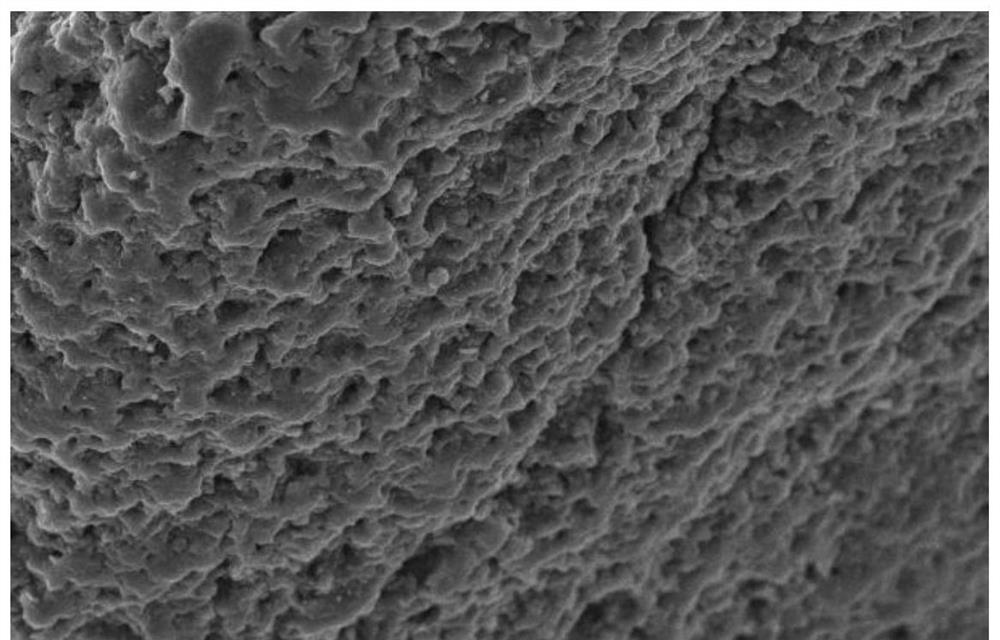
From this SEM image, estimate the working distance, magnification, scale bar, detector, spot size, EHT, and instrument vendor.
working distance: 13 mm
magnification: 0.2 K X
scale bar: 100000 nm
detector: SEI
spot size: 15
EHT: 30 kV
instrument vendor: JEOL